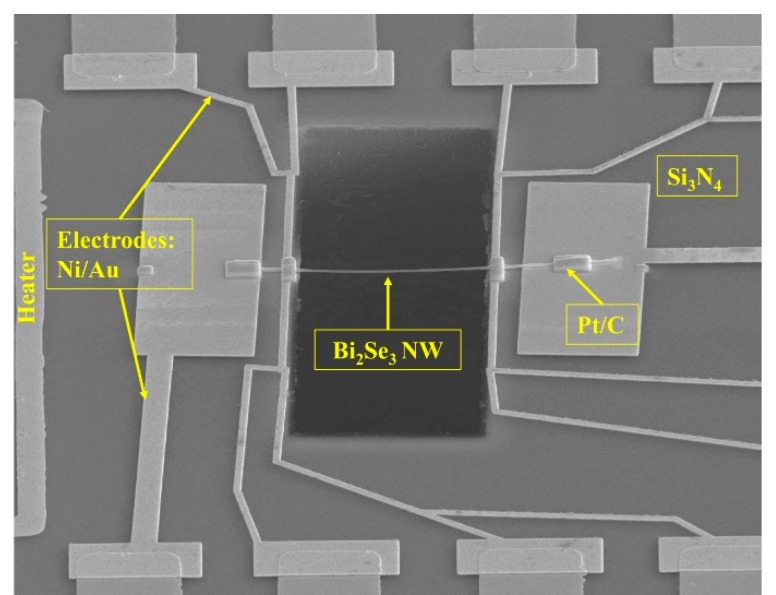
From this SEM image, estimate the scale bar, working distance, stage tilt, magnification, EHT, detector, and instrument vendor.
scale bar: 20000 nm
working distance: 5.4 mm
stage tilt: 52°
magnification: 2 K X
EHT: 15 kV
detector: ETD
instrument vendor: FEI